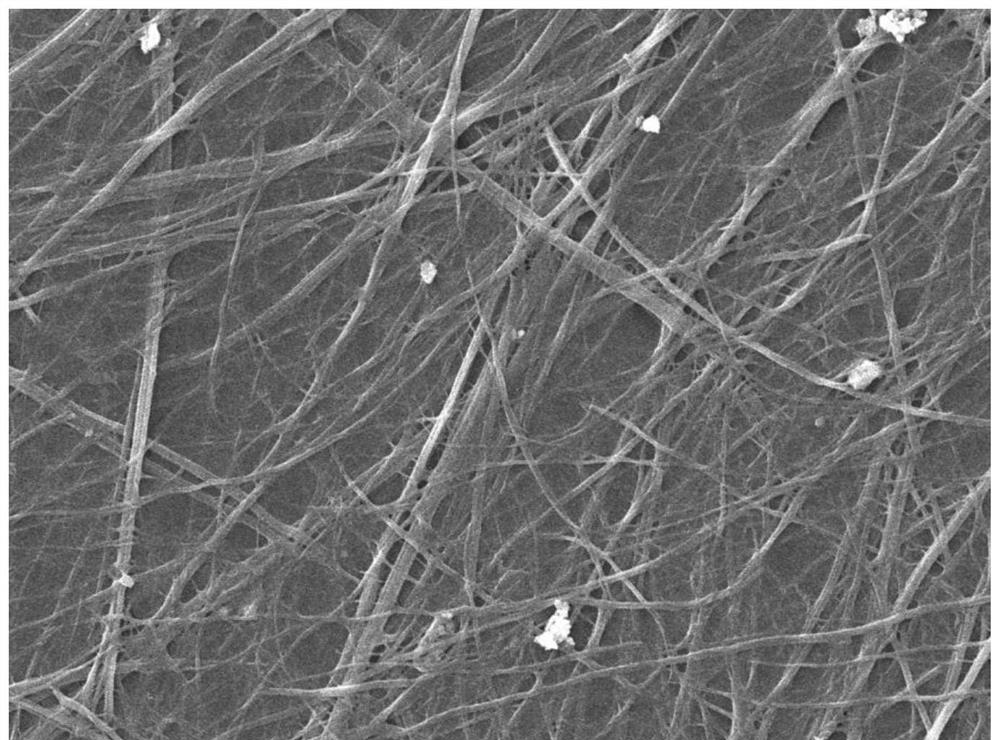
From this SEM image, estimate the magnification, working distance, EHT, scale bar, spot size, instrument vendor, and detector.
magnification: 1 K X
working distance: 13.2 mm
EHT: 20 kV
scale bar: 10000 nm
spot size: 30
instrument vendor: JEOL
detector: SED